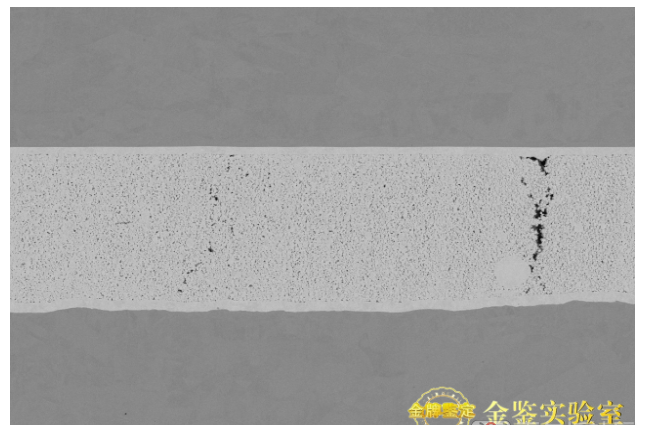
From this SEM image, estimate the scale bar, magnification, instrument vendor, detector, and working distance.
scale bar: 100000 nm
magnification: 1 K X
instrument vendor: Hitachi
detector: NL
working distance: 7.5 mm